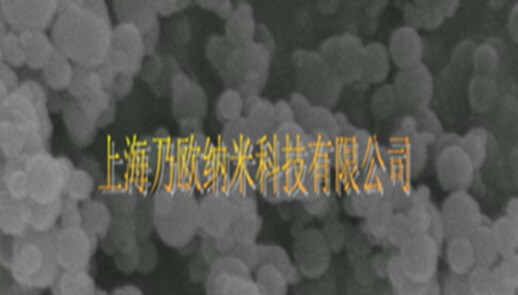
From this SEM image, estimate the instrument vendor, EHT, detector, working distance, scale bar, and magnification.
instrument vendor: FEI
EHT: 20 kV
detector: ETD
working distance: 9.7 mm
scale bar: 100 nm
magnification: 10 K X